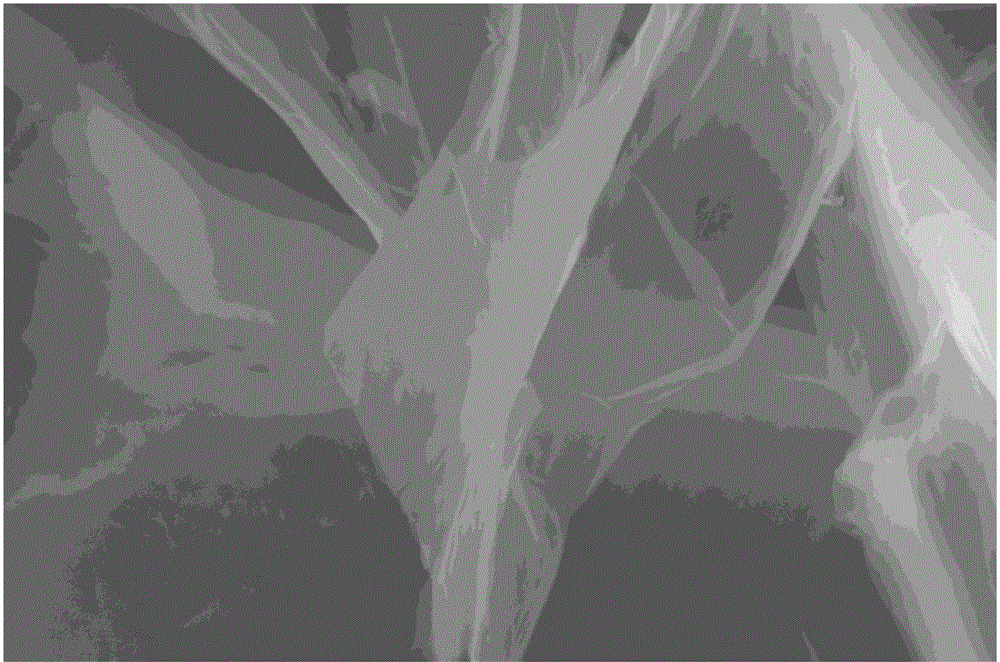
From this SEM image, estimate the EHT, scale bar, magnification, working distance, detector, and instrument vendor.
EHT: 20 kV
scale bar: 10000 nm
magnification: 20 K X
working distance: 5.8 mm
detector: ETD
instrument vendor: FEI